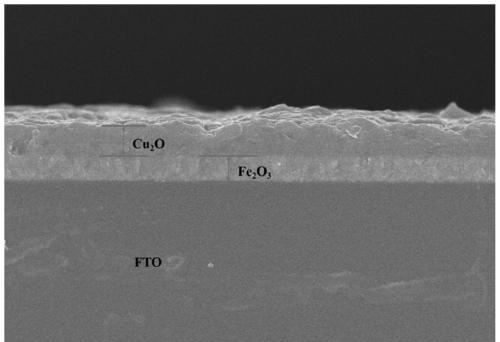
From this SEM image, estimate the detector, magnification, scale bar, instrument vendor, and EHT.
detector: SE(U)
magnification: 10 K X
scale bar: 5000 nm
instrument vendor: Hitachi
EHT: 10 kV